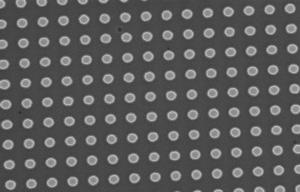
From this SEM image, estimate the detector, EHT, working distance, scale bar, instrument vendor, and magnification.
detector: InLens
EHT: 10 kV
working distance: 7 mm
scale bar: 1000 nm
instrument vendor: Zeiss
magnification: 9.53 K X